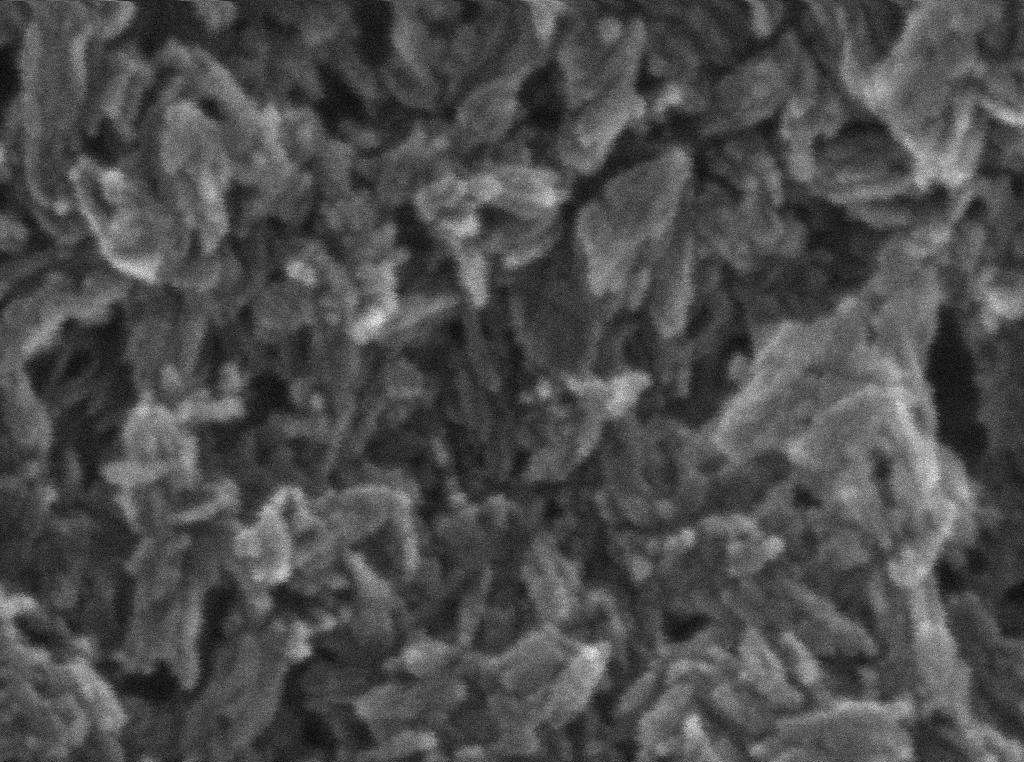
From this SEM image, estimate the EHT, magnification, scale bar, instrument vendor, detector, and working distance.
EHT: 20 kV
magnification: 400 K X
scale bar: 200 nm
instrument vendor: Tescan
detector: InBeam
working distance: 4.652 mm